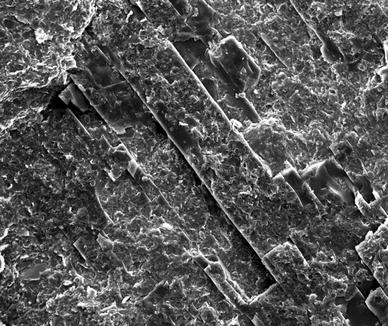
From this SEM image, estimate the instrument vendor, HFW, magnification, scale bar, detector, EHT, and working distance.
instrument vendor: FEI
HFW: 512 µm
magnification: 0.5 K X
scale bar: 200000 nm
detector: ETD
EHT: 20 kV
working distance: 13.5 mm